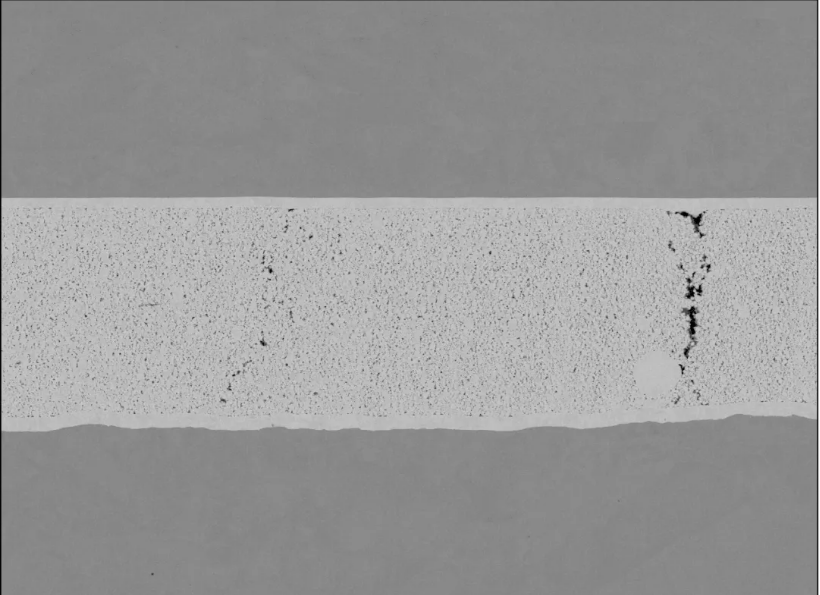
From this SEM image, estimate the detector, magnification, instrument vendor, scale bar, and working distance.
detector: NL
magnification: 1 K X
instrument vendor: Hitachi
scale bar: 100000 nm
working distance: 7.5 mm